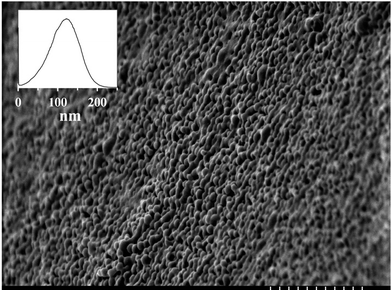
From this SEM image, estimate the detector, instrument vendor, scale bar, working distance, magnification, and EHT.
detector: SE(M)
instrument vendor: Hitachi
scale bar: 1000 nm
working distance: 8 mm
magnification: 30 K X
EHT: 1.5 kV